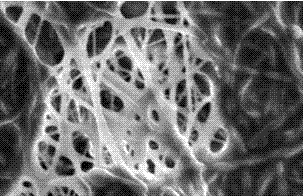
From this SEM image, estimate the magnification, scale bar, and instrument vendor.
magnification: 5 K X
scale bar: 5000 nm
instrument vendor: JEOL